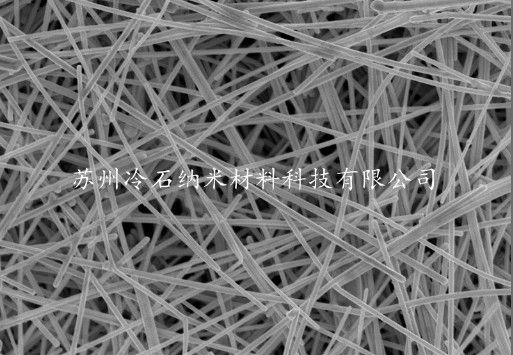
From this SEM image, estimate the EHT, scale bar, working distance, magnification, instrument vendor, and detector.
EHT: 5 kV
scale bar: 2000 nm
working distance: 8.3 mm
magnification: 20 K X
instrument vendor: Hitachi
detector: SE(M)